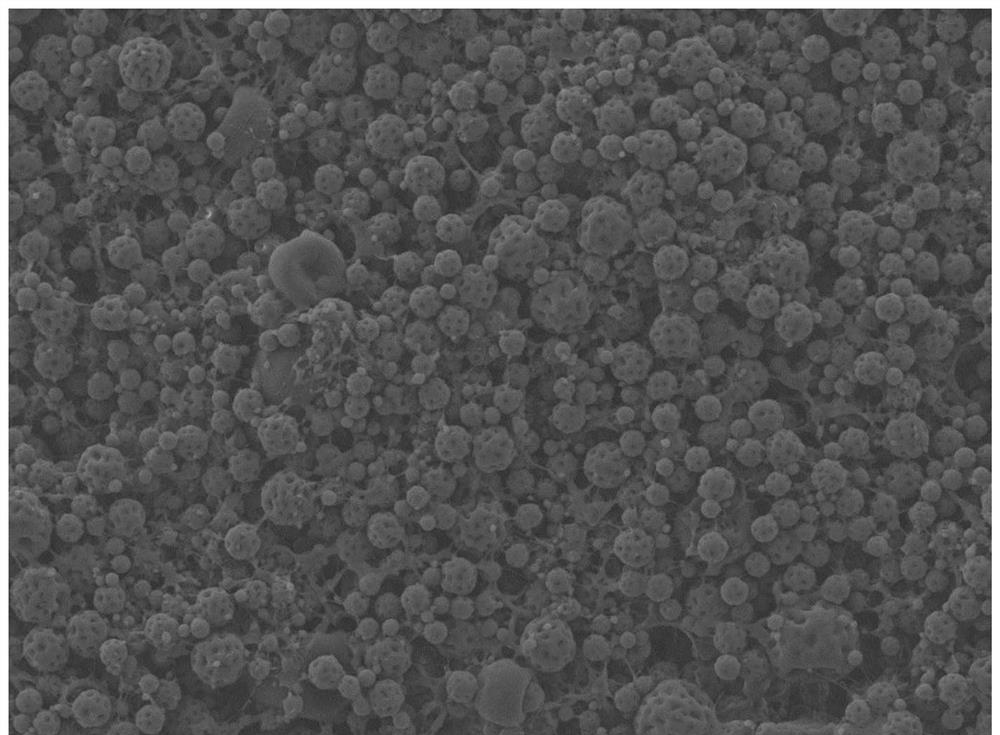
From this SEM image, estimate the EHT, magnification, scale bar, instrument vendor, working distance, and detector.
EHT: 5 kV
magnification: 2 K X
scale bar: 10000 nm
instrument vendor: JEOL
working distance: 8 mm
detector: LED